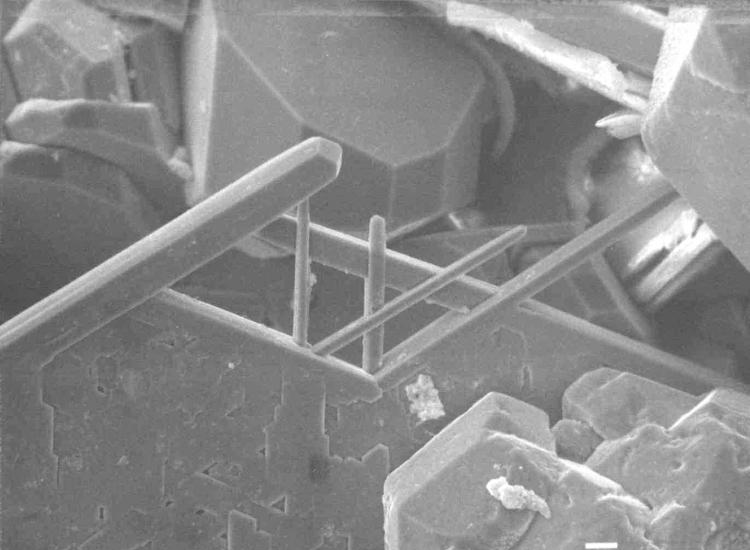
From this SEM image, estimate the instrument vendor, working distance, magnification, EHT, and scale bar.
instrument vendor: JEOL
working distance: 35 mm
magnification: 0.5 K X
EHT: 20 kV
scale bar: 10000 nm